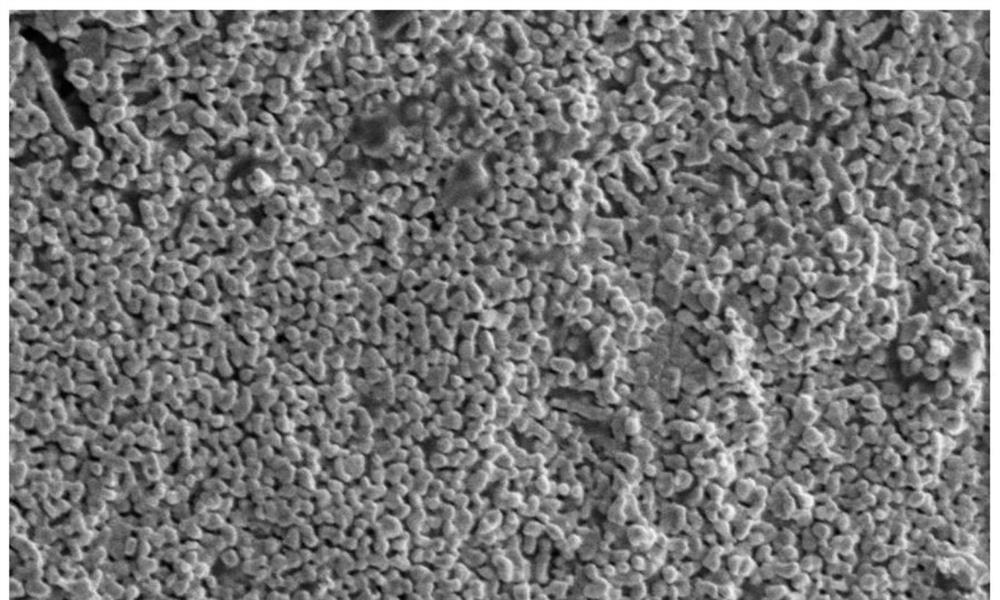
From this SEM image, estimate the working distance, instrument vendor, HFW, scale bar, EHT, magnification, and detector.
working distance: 9.6 mm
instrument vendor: FEI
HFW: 20.7 µm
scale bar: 5000 nm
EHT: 5 kV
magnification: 20 K X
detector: ETD